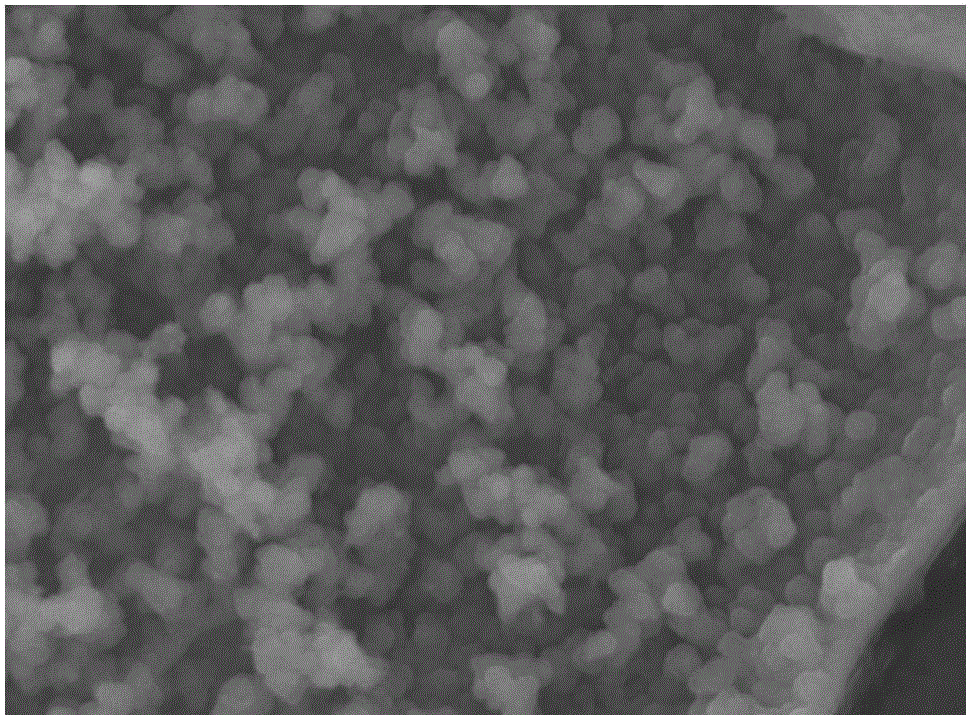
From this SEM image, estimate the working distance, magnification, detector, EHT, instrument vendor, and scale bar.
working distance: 10.6 mm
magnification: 5 K X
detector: SED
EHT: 20 kV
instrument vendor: JEOL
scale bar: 5000 nm